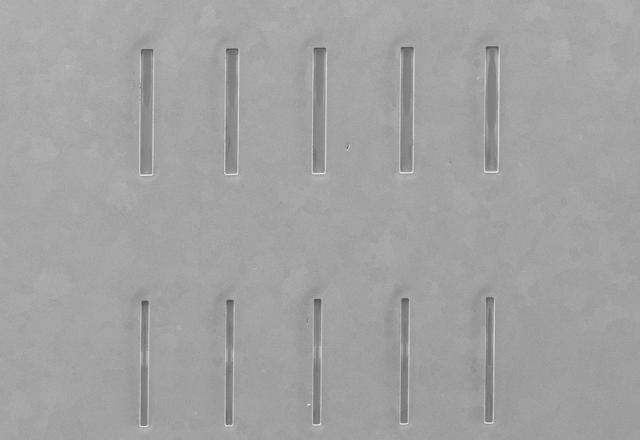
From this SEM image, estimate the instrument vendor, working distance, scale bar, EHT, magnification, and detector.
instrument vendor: Hitachi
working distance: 12.2 mm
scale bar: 1000000 nm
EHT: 5 kV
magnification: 0.05 K X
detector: SE(M)